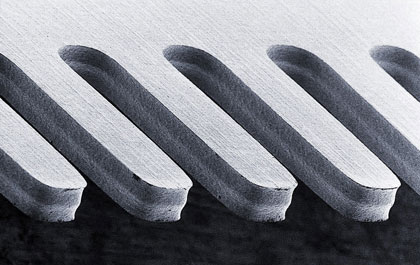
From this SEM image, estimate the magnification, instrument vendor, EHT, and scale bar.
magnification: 0.075 K X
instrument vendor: other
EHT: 5 kV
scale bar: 100000 nm